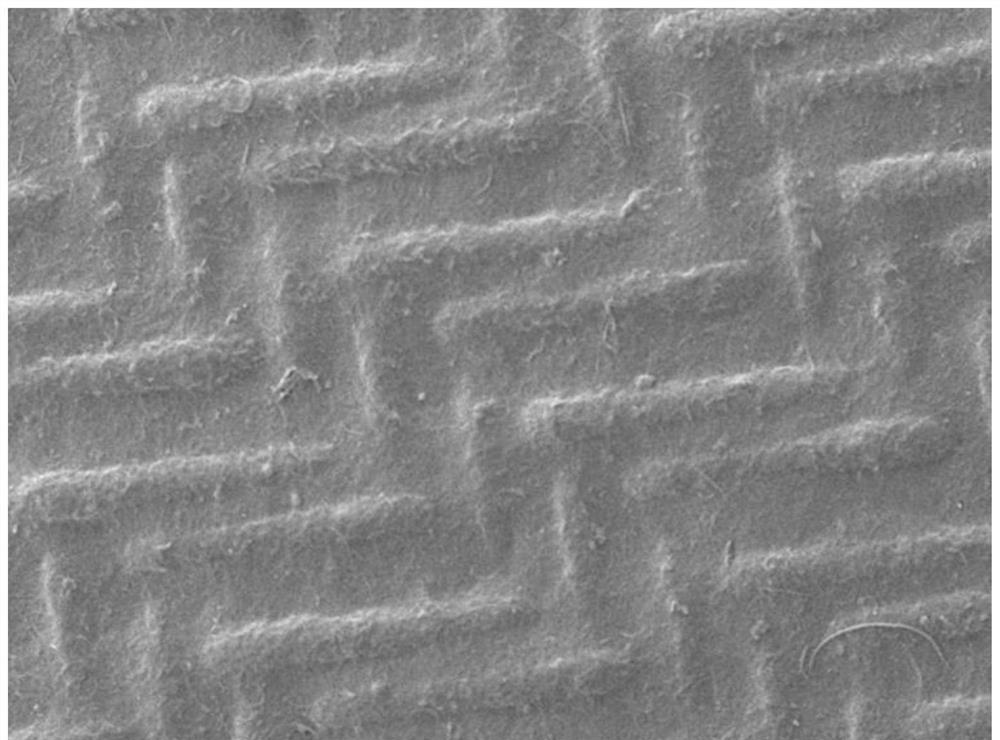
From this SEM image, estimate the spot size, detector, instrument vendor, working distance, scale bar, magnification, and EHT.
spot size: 30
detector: SED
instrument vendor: JEOL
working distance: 11.3 mm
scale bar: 100000 nm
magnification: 0.1 K X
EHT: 15 kV